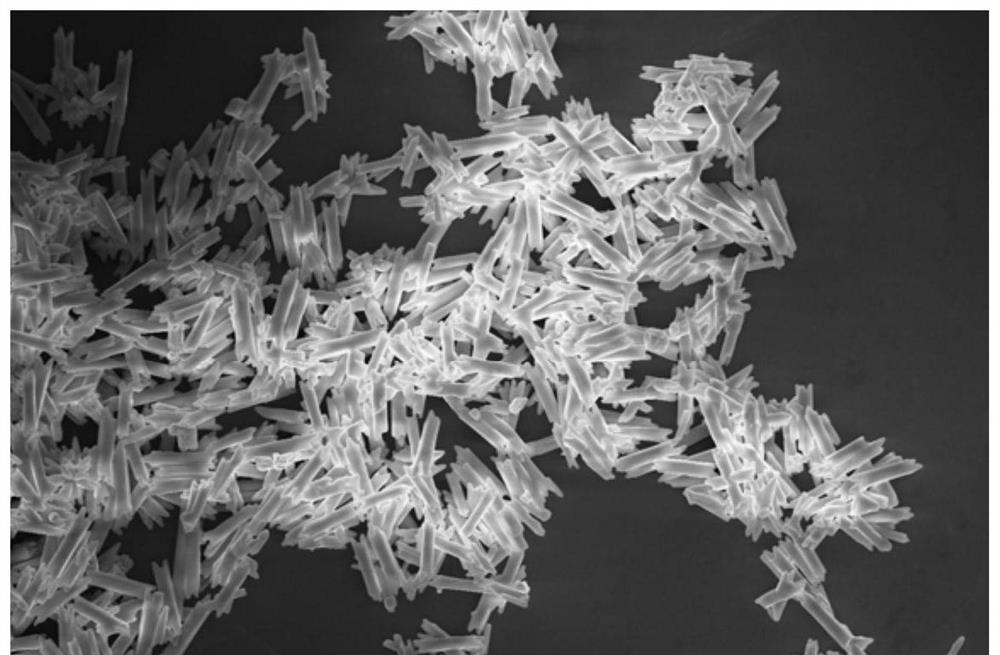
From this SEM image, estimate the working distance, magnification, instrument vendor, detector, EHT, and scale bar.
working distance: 3.6 mm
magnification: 3.83 K X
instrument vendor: FEI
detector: TLD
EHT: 10 kV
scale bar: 40000 nm